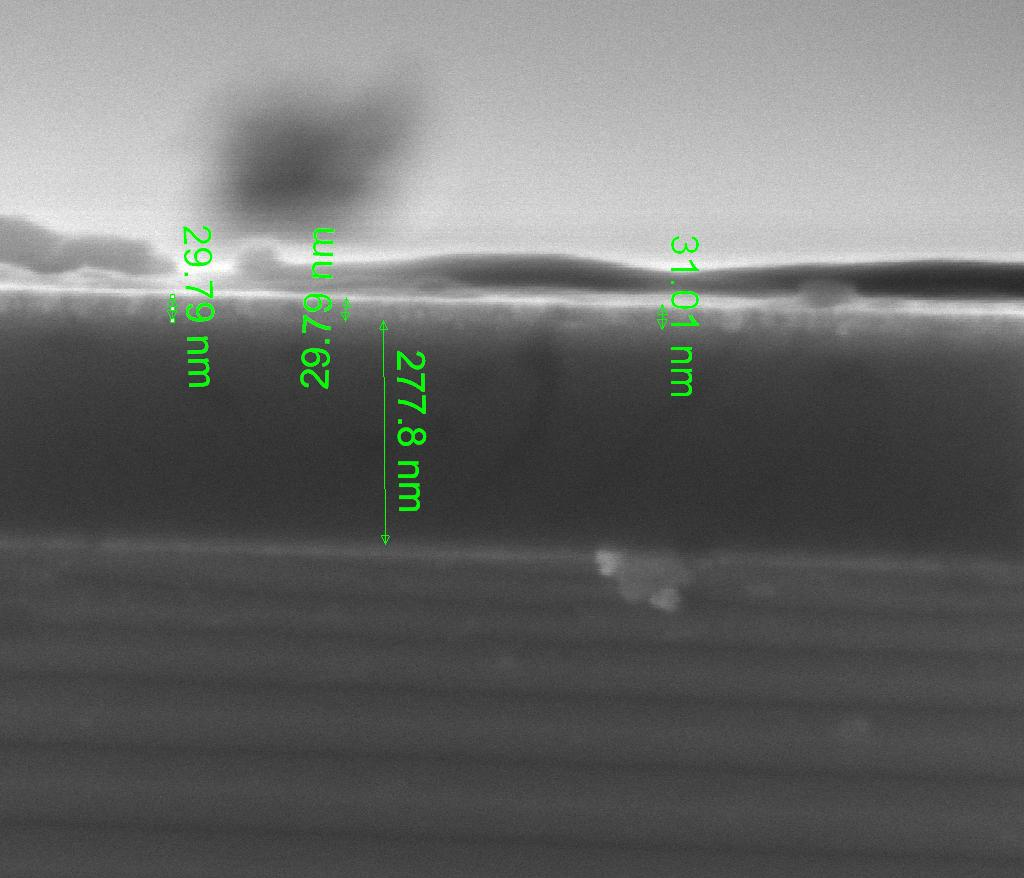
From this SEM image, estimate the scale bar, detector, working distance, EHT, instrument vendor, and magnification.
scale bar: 300 nm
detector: TLD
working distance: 5.7 mm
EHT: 8 kV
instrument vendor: FEI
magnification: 100 K X